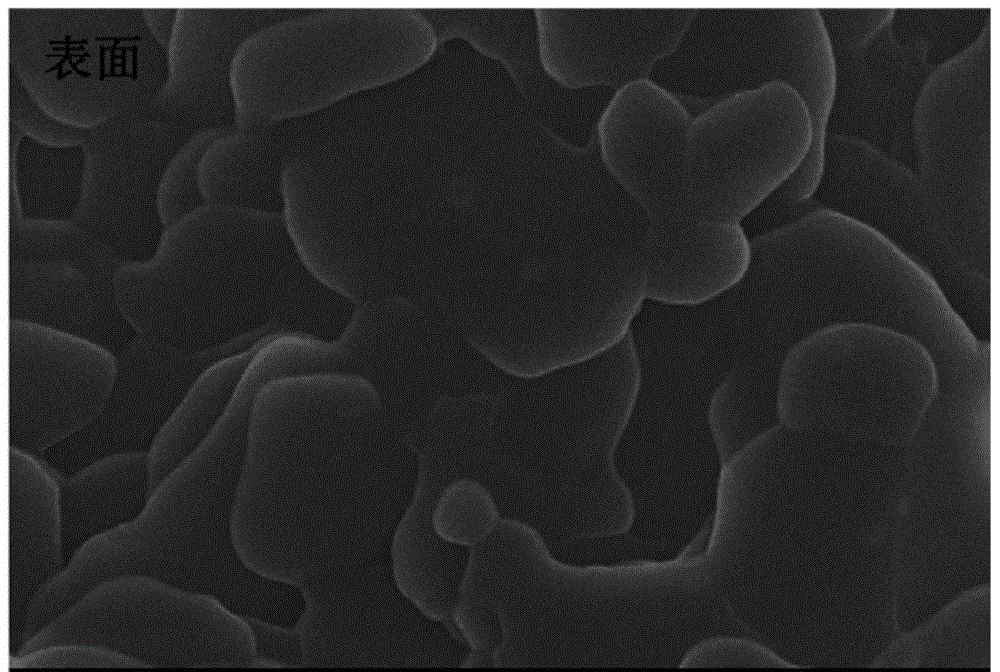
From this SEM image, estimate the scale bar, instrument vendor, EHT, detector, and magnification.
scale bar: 1000 nm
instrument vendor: JEOL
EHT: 20 kV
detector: SEI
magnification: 10 K X